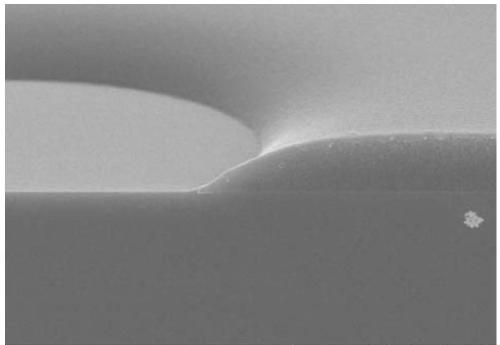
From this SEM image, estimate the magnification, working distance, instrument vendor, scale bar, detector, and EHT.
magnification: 5 K X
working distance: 7.7 mm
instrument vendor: Hitachi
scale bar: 10000 nm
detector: SE(UL)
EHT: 15 kV